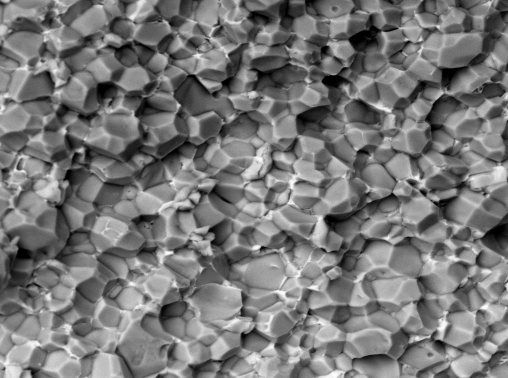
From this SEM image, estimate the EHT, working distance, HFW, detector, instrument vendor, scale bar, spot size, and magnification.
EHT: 20 kV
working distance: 7.69 mm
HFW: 70.27 µm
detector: BSE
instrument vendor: FEI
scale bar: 5000 nm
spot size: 1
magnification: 5 K X